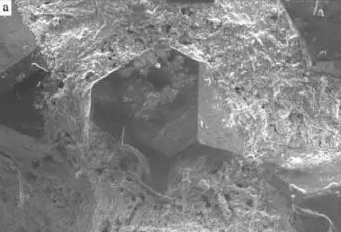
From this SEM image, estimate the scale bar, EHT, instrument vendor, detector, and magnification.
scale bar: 300000 nm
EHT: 20 kV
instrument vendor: other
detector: SED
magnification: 0.15 K X